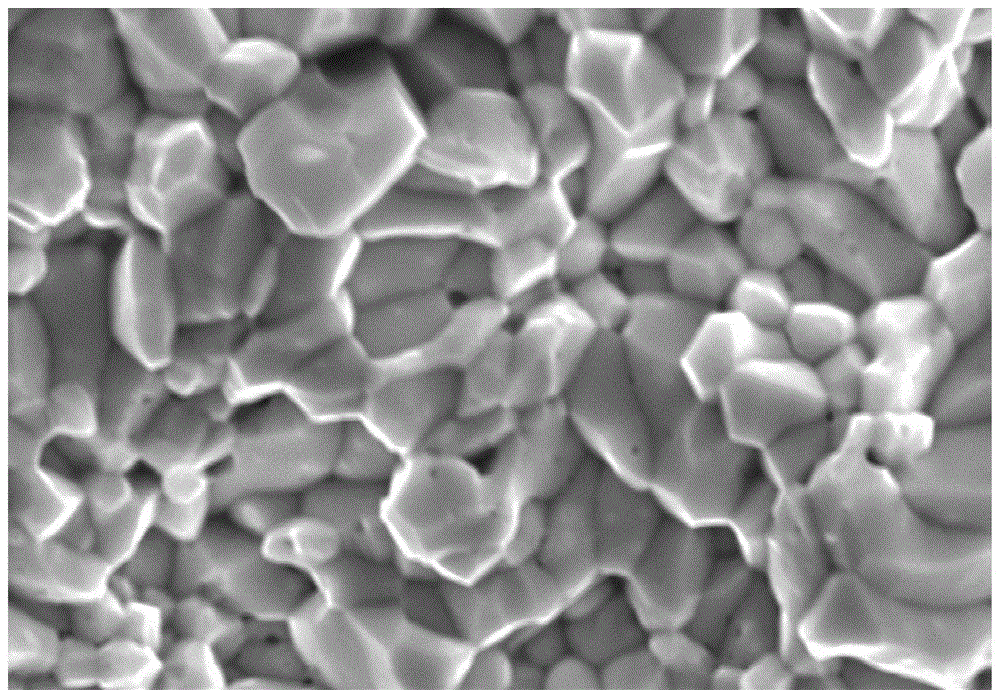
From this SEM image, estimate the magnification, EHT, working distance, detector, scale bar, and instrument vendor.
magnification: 20 K X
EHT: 10 kV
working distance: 15 mm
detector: SE(UL)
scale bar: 2000 nm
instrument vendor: Hitachi